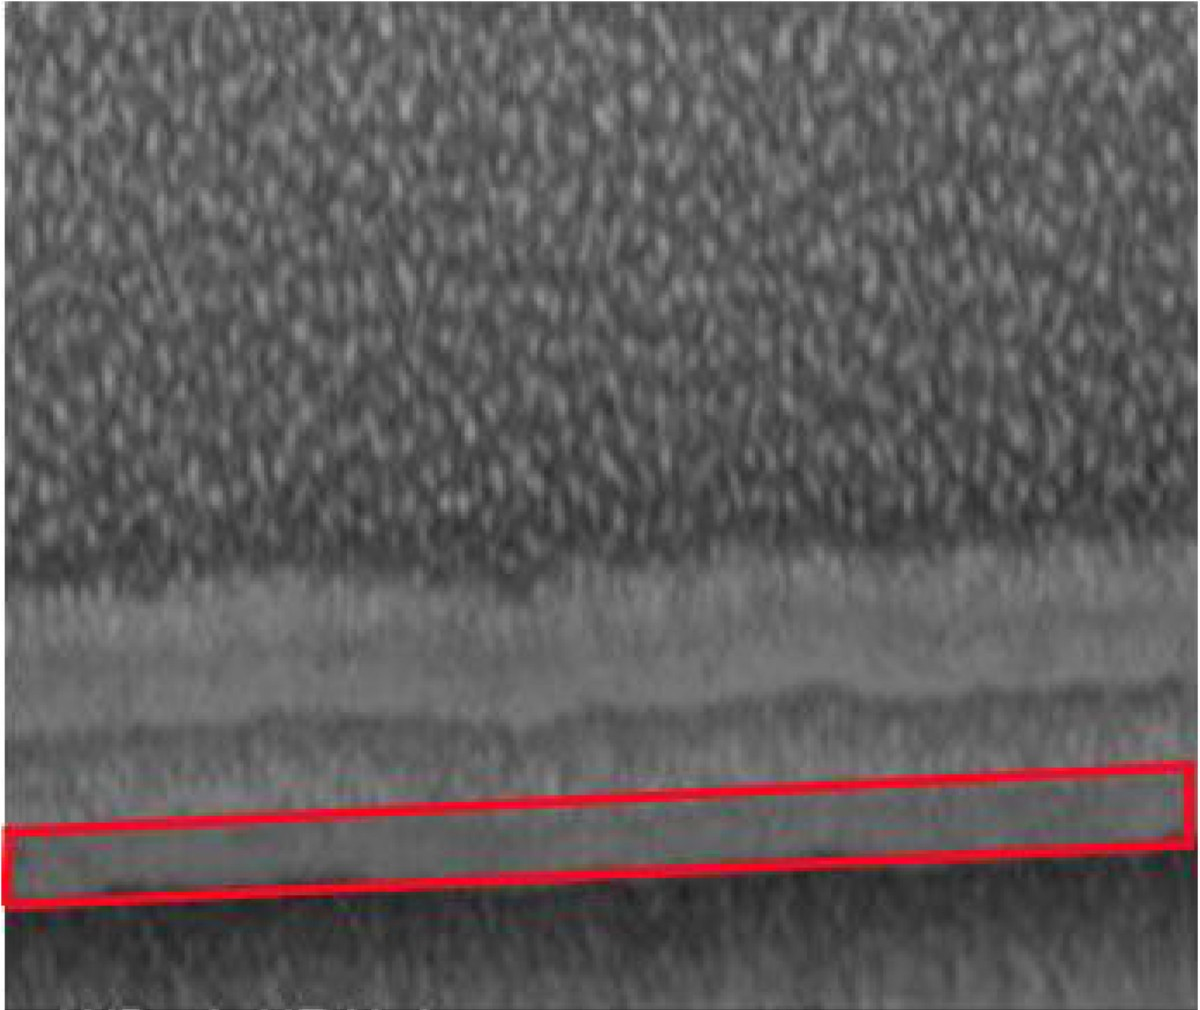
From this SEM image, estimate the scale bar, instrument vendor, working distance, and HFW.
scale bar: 400 nm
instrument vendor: FEI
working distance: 4.1 mm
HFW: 1.28 µm